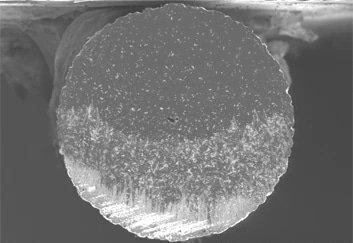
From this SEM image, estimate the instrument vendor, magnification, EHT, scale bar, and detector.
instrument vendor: Hitachi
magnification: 0.15 K X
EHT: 5 kV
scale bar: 300000 nm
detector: SE(U)